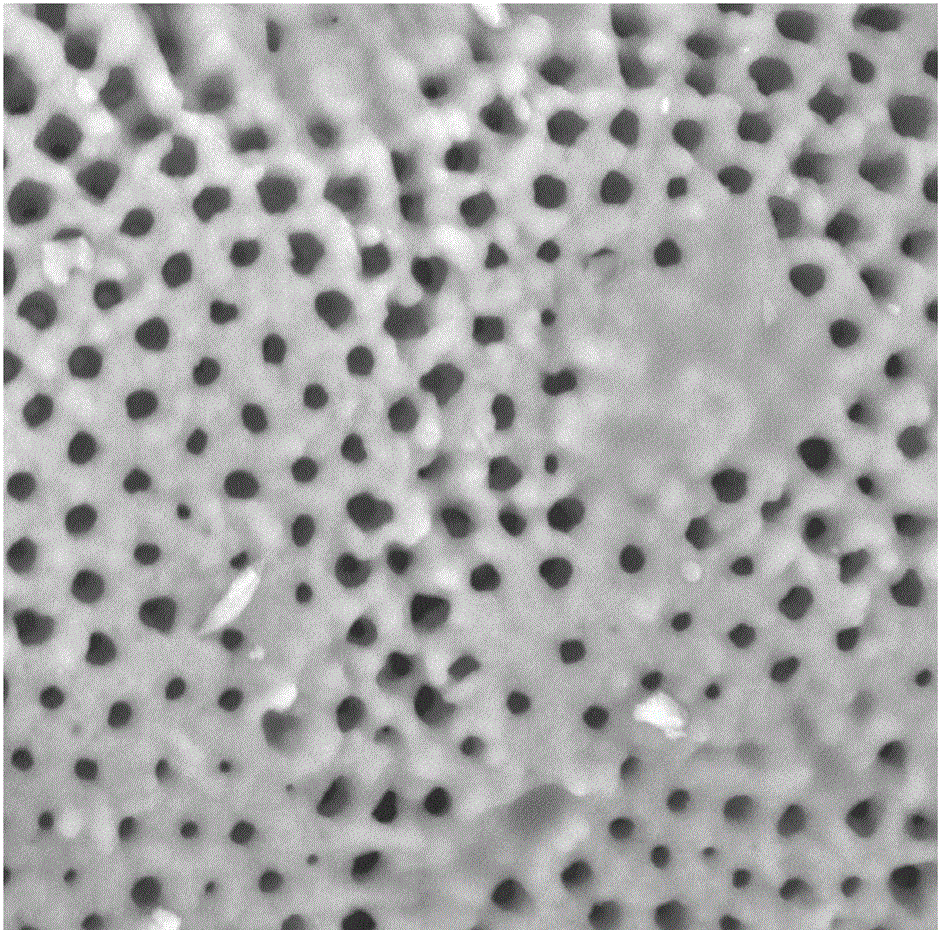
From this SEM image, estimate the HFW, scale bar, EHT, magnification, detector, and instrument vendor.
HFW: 13.5 µm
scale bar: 3000 nm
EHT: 10 kV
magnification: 20 K X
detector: BSD Full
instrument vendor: Thermo